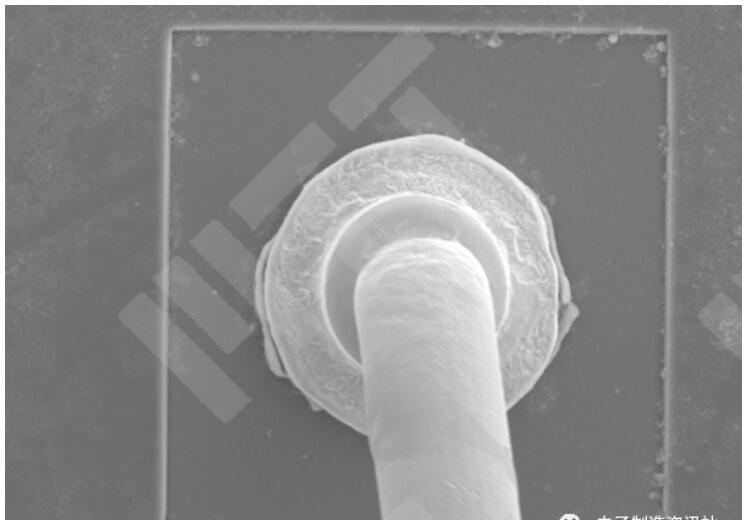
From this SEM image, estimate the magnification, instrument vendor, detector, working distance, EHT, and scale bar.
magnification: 1 K X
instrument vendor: Hitachi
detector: SE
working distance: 20.1 mm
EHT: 15 kV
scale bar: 50000 nm